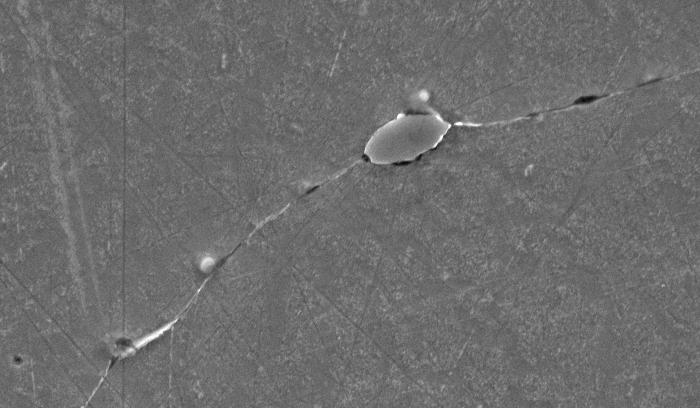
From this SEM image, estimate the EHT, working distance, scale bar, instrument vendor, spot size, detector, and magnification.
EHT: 20 kV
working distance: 11.2 mm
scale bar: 10000 nm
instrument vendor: FEI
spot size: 3.6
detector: SE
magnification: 1.5 K X